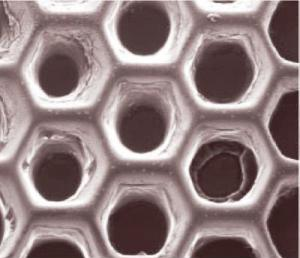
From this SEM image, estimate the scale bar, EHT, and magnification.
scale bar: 500000 nm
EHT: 15 kV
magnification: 0.063 K X